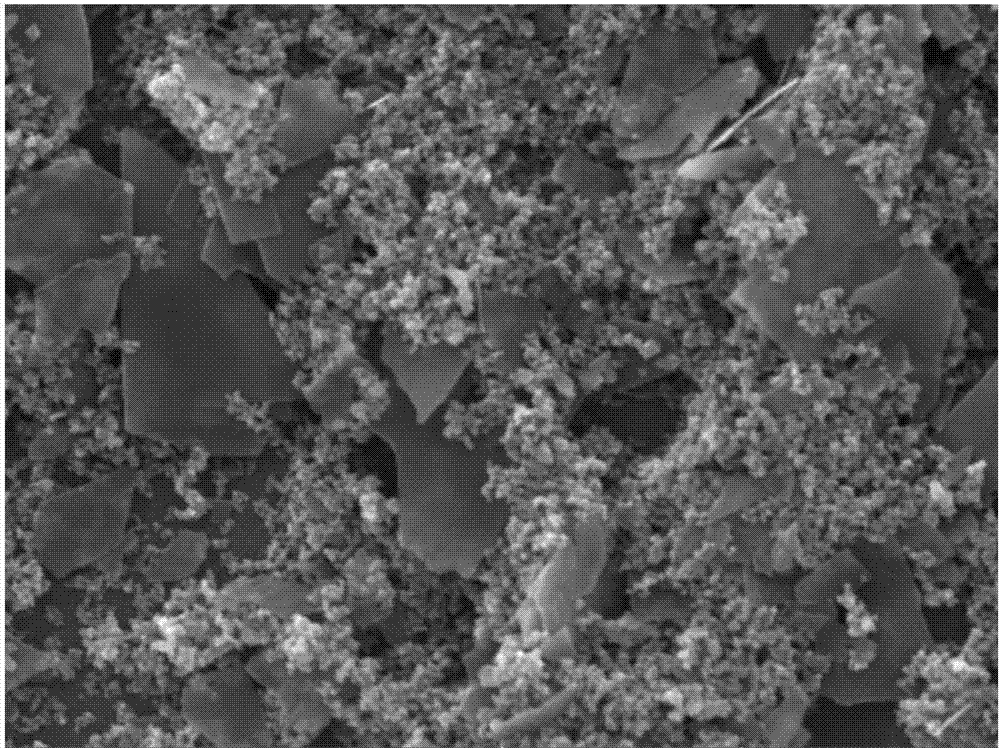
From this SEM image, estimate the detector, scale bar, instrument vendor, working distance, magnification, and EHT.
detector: SE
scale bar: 5000 nm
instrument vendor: Tescan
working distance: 8.37 mm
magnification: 10 K X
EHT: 30 kV